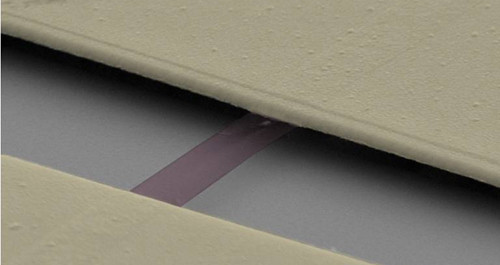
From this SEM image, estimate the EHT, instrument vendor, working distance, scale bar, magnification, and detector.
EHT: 10 kV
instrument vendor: Zeiss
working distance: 7 mm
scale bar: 200 nm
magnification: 75.58 K X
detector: SE2